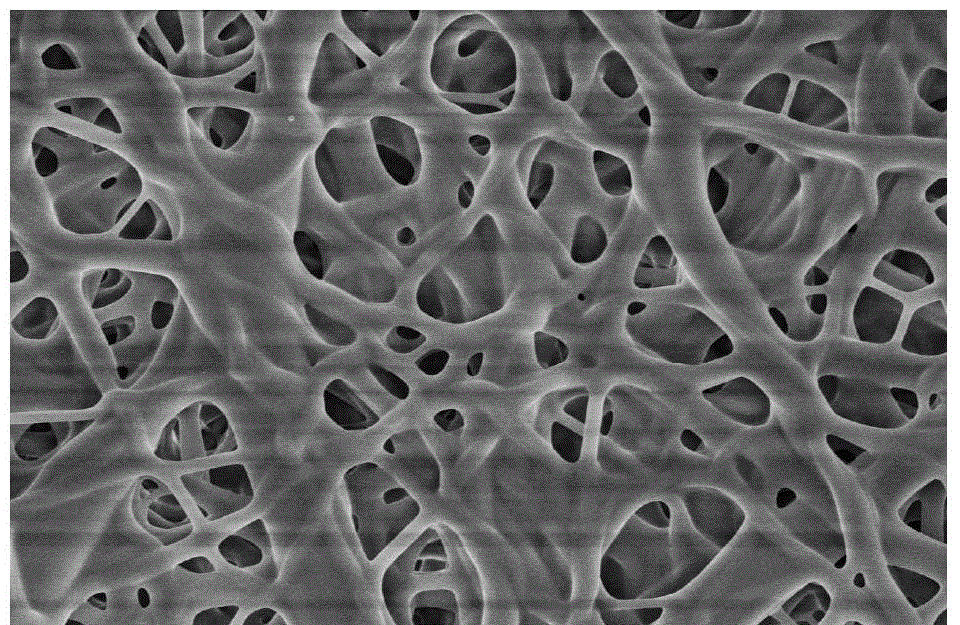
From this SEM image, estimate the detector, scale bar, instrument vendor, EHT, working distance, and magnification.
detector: SE(M)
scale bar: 10000 nm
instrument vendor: Hitachi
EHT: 5 kV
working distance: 8.7 mm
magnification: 5 K X